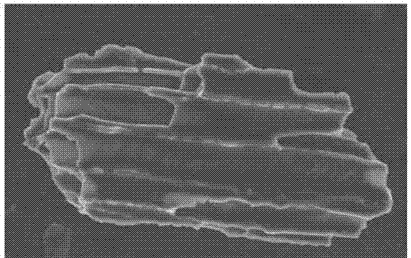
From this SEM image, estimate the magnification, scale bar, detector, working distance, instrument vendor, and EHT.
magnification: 1.13 K X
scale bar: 10000 nm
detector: InLens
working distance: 9.3 mm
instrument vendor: Zeiss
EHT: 10 kV